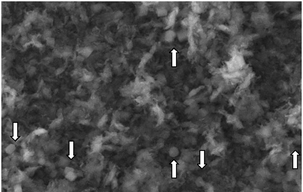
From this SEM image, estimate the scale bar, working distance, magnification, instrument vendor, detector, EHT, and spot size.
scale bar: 5000 nm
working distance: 12.2 mm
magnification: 5 K X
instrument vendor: FEI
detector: SE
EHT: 20 kV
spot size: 3